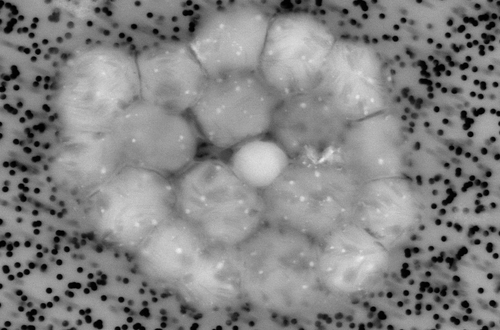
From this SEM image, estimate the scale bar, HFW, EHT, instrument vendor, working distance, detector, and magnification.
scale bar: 2000 nm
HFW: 20.65 µm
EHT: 15 kV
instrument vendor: Zeiss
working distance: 7.3 mm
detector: AsB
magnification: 5.54 K X